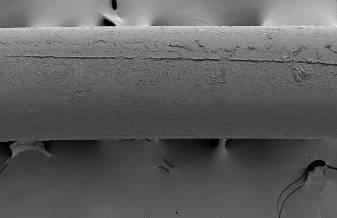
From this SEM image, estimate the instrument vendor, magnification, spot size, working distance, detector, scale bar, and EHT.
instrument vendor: FEI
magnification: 0.25 K X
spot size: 3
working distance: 5.6 mm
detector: SE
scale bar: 200000 nm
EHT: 1 kV